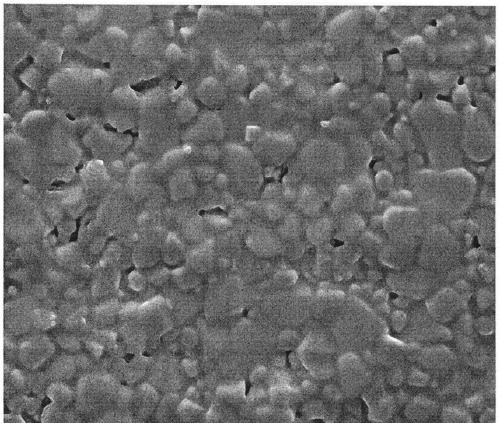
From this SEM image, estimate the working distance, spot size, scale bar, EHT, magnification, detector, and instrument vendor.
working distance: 4.1 mm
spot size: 3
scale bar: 10000 nm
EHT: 10 kV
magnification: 5 K X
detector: ETD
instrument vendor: FEI